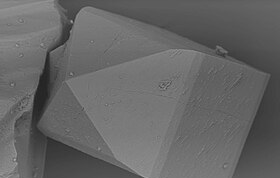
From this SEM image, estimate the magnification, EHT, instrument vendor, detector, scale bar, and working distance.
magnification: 2 K X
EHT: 15 kV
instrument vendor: Zeiss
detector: QBSD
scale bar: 10000 nm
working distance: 11 mm